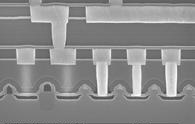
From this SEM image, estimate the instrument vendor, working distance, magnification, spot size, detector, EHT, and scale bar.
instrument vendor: FEI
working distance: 6.1 mm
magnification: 30 K X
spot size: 3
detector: TLD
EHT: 10 kV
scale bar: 1000 nm